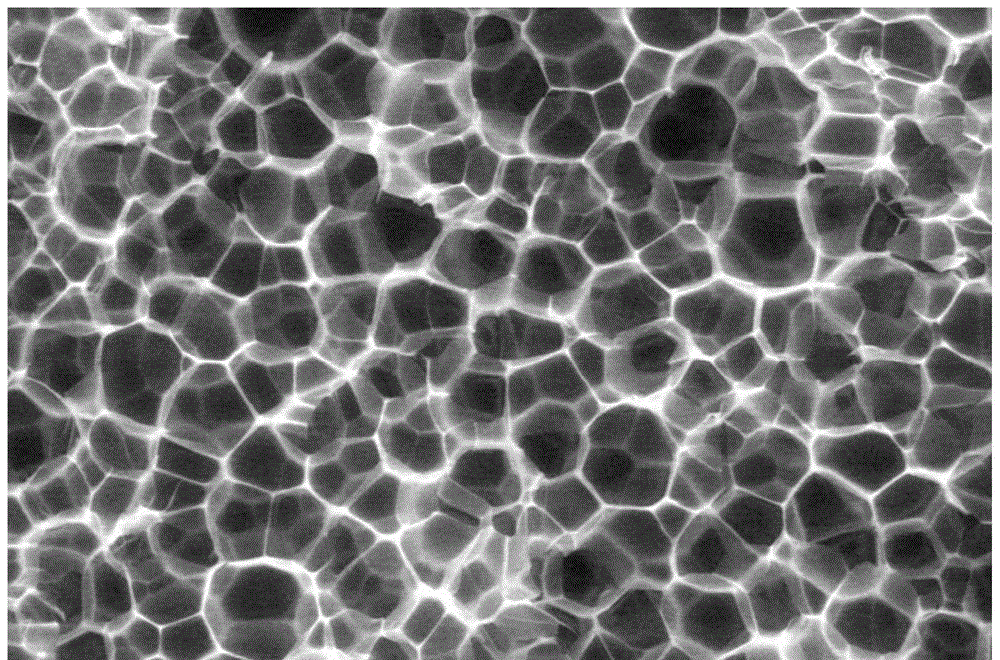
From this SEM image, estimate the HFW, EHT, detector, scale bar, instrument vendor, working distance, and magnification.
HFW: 518 µm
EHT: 20 kV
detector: LFD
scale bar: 100000 nm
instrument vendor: FEI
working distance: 65.5 mm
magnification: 0.8 K X